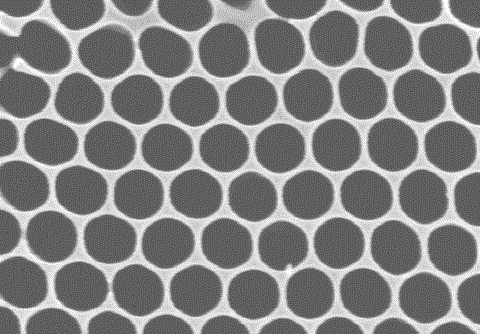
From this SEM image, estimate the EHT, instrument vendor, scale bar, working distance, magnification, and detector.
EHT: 10 kV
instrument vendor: Hitachi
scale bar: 300 nm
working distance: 9.8 mm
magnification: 150 K X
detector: SE(M)